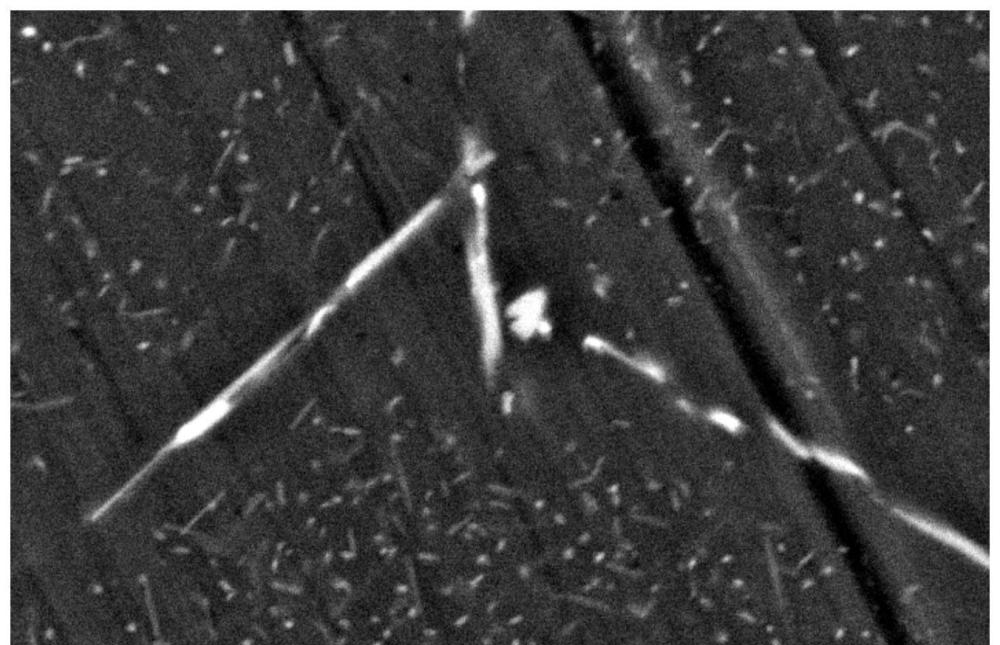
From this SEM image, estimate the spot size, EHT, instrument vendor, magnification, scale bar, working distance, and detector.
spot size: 5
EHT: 20 kV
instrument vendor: FEI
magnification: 10 K X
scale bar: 5000 nm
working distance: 6.2 mm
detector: BSE